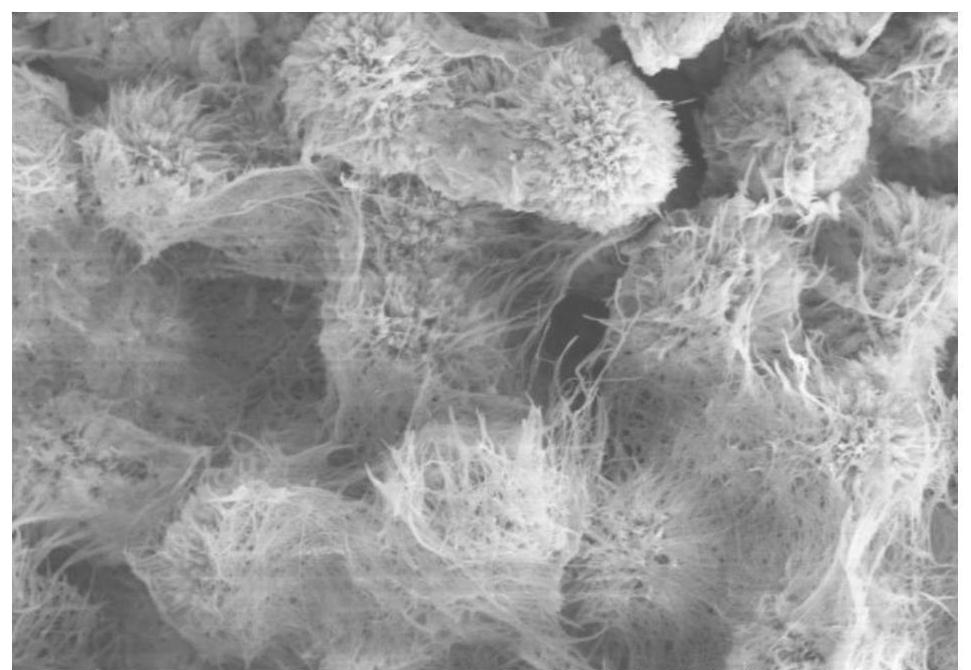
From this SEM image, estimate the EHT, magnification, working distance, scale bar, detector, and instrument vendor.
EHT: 5 kV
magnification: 10 K X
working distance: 19.1 mm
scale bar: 5000 nm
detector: SE(M)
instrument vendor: Hitachi